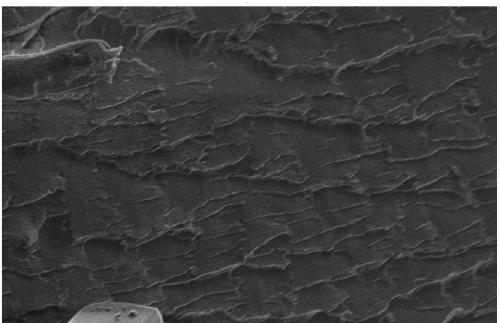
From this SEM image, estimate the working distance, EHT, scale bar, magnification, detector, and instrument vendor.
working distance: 9.5 mm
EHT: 5 kV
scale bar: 10000 nm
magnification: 1 K X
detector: SE1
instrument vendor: Zeiss